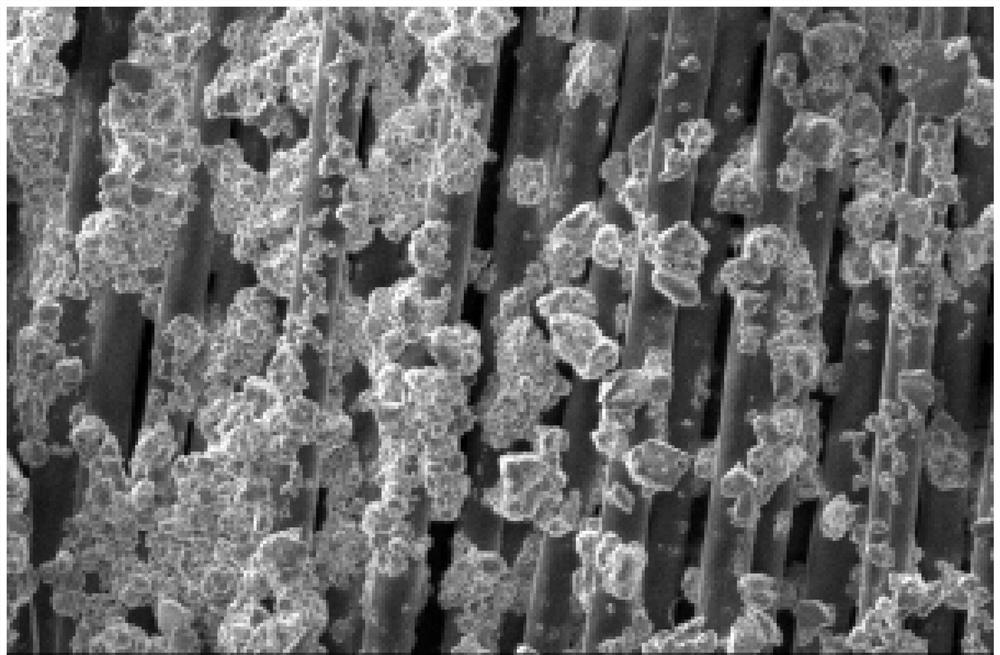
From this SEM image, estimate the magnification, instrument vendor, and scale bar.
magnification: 0.5 K X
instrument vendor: JEOL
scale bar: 50000 nm